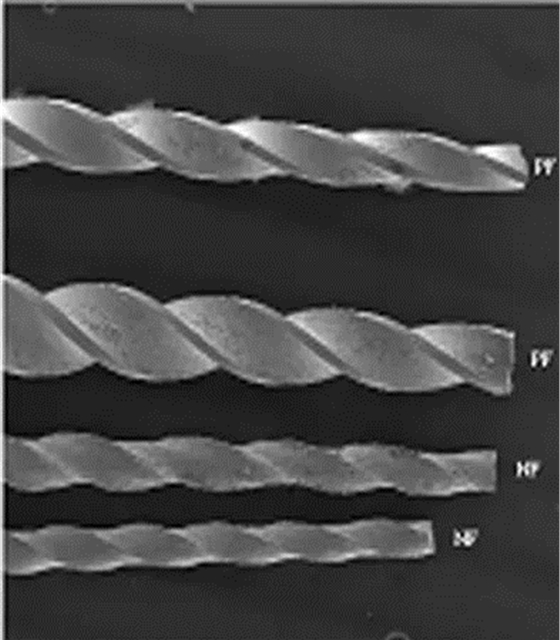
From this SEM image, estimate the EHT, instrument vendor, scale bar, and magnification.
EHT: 20 kV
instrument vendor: JEOL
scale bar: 1000000 nm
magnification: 0.03 K X